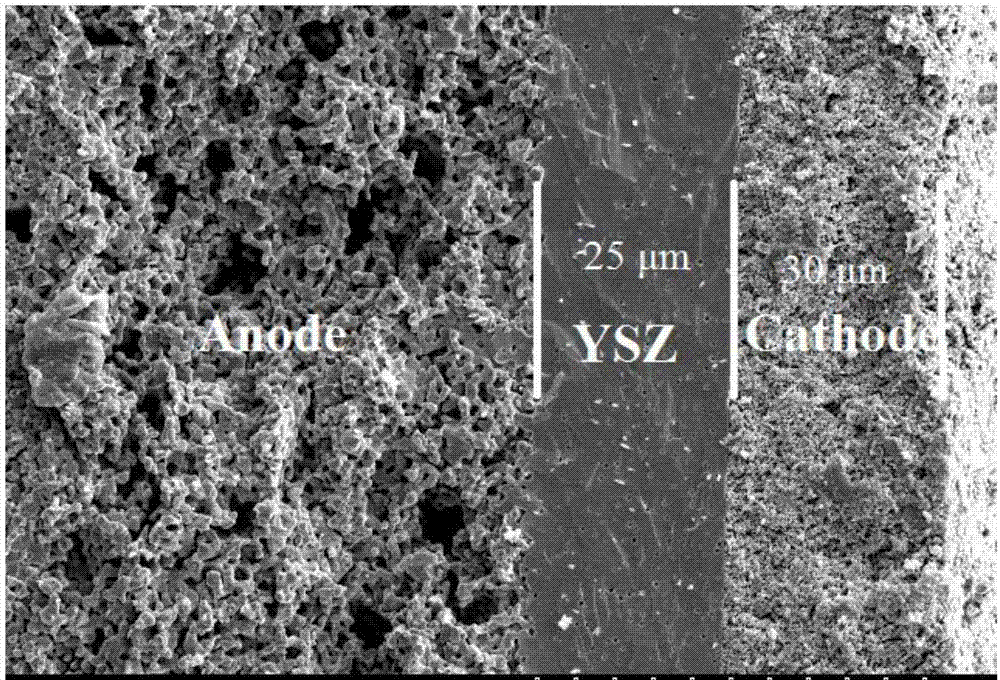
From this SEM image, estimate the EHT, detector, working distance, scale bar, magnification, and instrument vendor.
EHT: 10 kV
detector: SE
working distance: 13.3 mm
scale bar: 50000 nm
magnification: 1 K X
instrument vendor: Hitachi